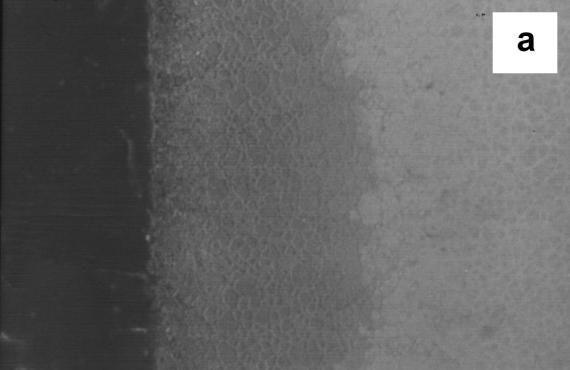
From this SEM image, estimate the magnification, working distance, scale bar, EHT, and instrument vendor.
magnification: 0.3 K X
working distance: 14 mm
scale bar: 100000 nm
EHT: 15 kV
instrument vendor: JEOL